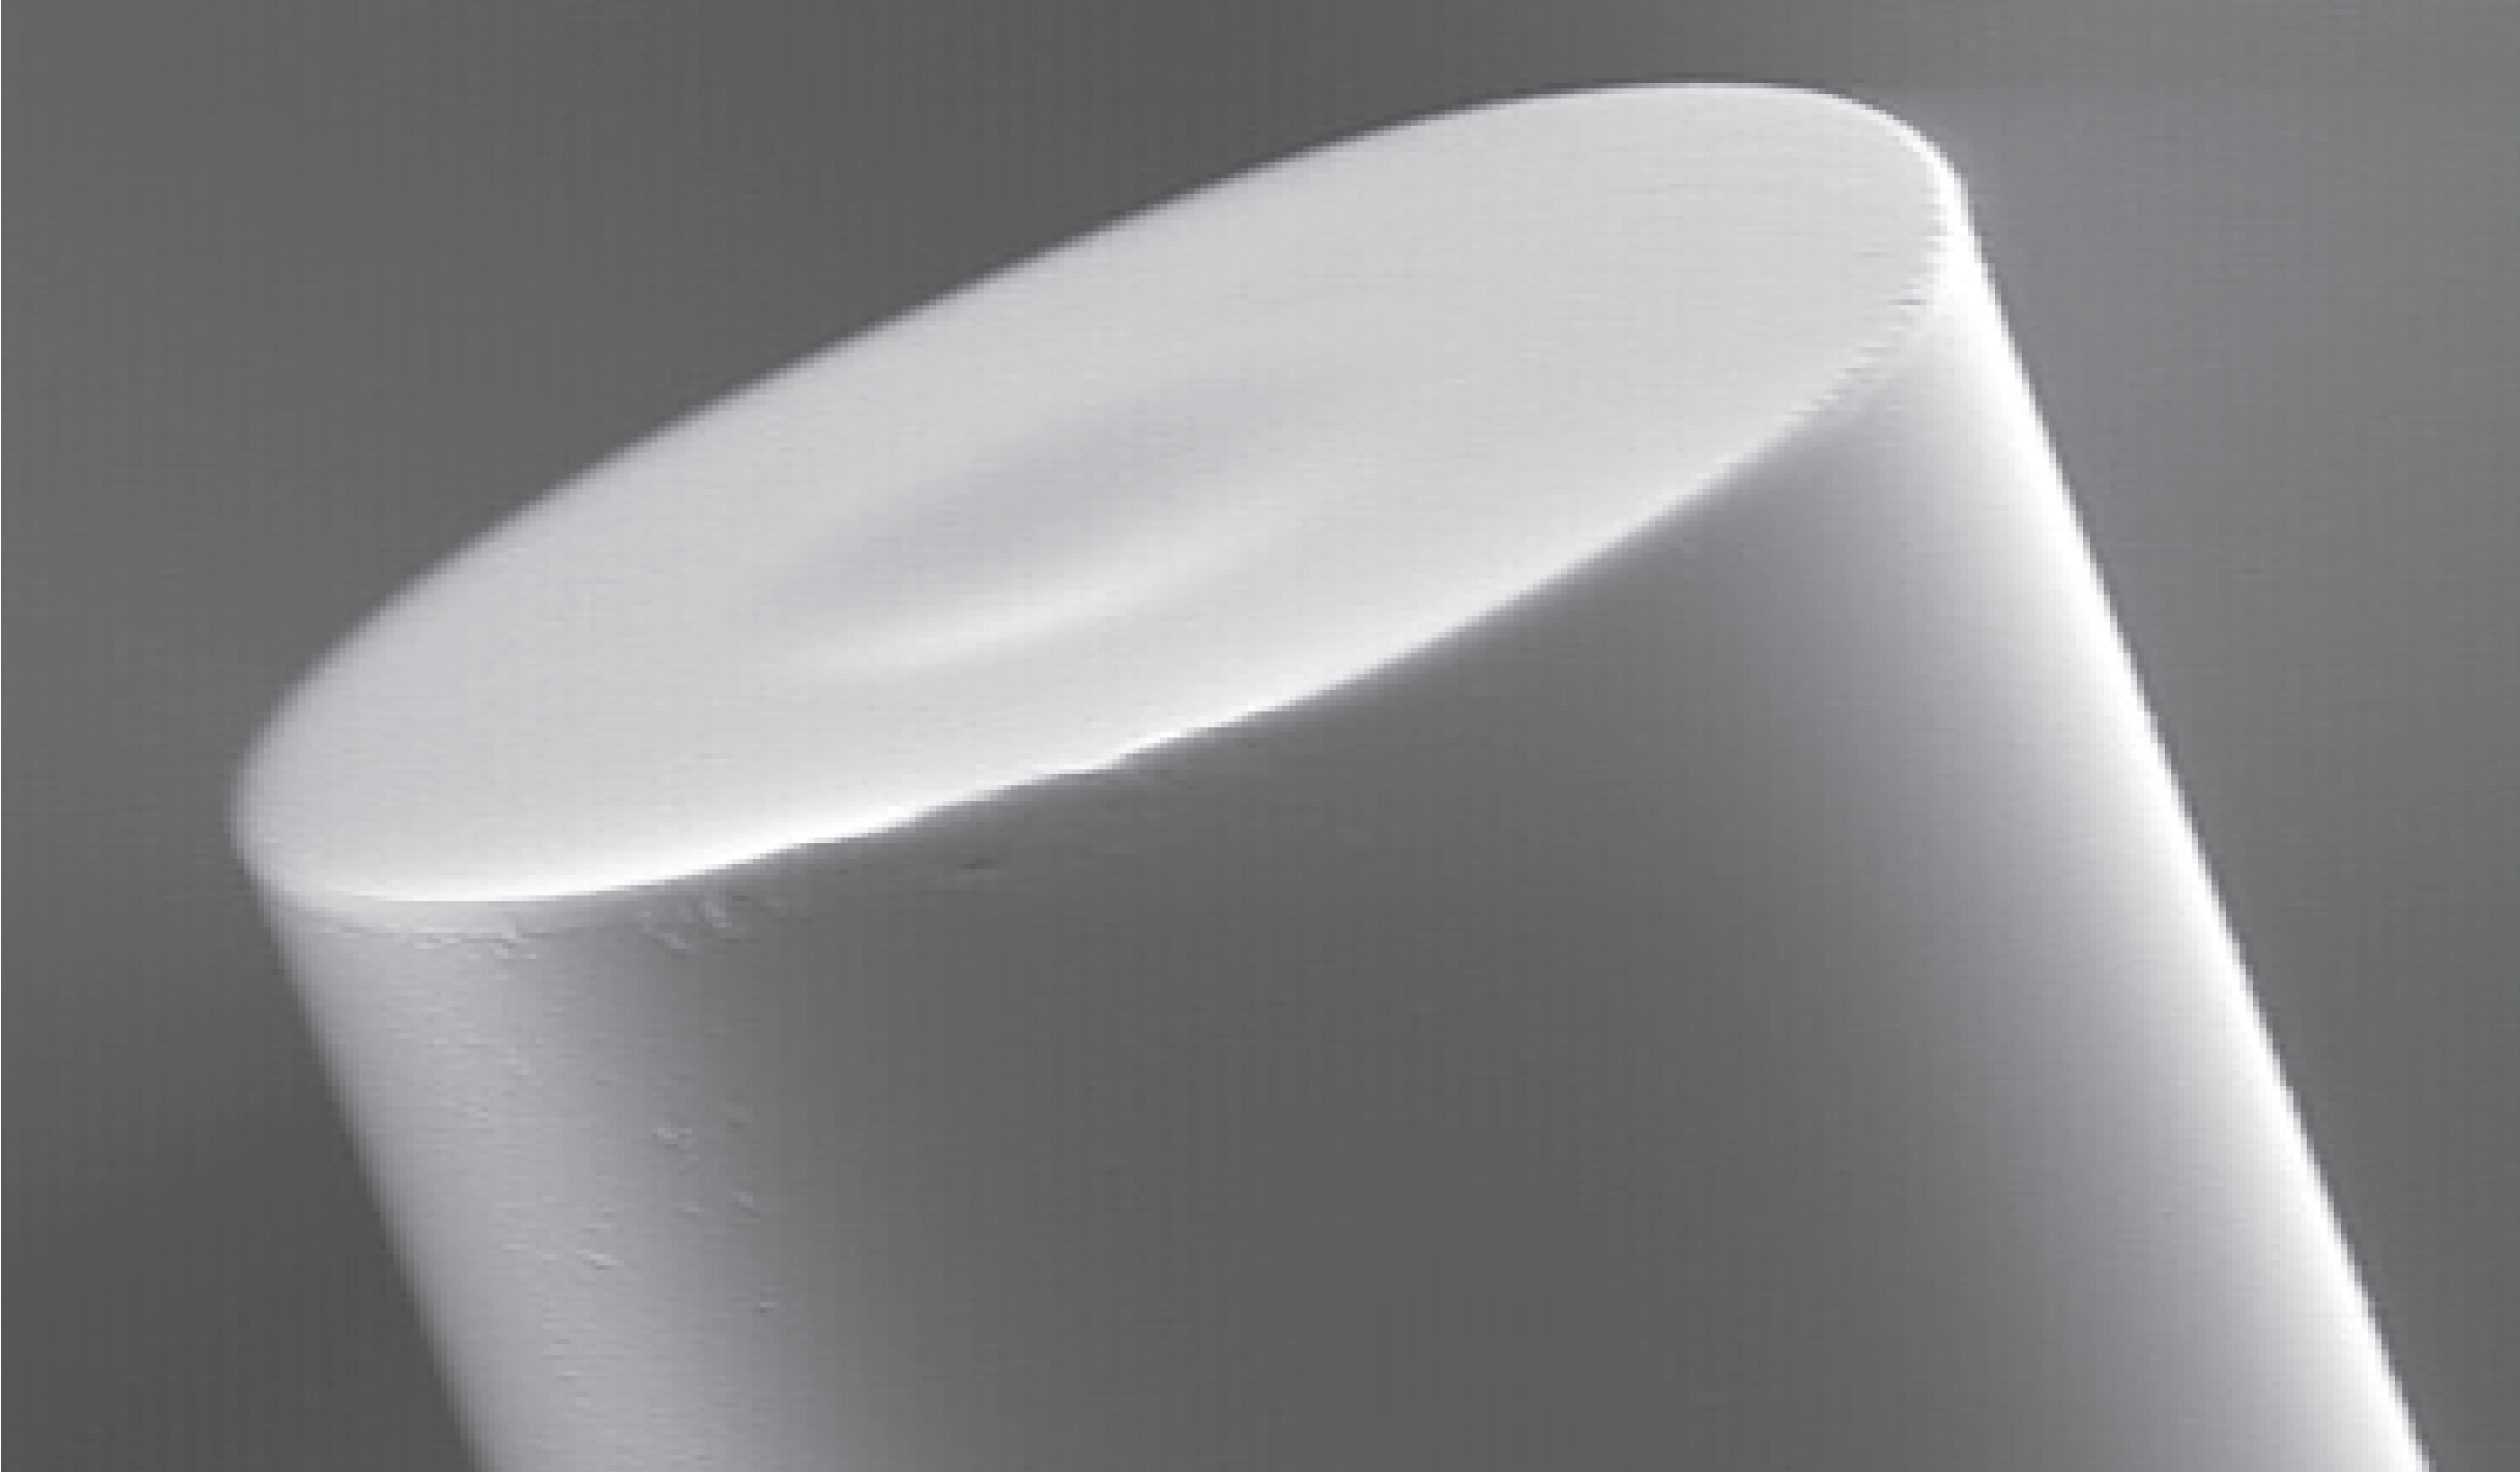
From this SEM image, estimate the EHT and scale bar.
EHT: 19 kV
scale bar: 50000 nm